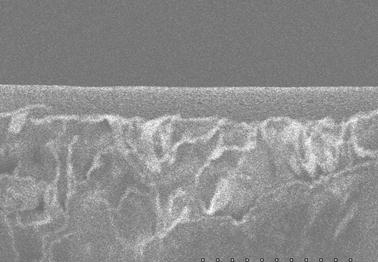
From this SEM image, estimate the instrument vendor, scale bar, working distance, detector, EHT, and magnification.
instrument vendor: JEOL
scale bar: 50000 nm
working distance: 12.2 mm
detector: SE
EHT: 5 kV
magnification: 1 K X